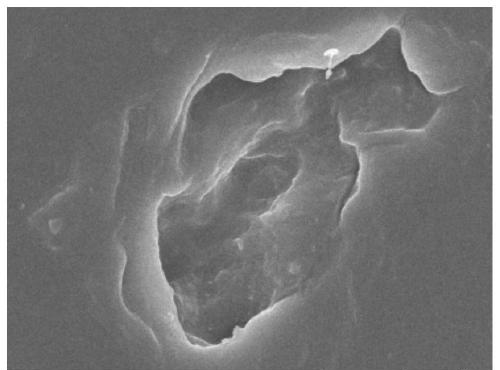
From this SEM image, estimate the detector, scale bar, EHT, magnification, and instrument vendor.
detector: SEI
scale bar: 1000 nm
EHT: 5 kV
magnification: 20 K X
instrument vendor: JEOL